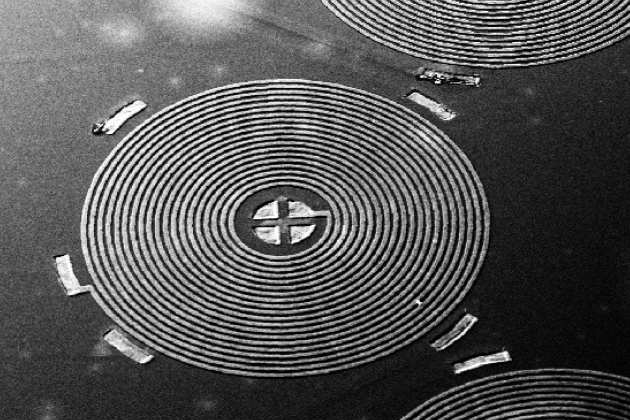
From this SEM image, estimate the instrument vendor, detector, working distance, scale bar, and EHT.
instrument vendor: Zeiss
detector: SE1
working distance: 29 mm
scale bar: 200000 nm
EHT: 15 kV